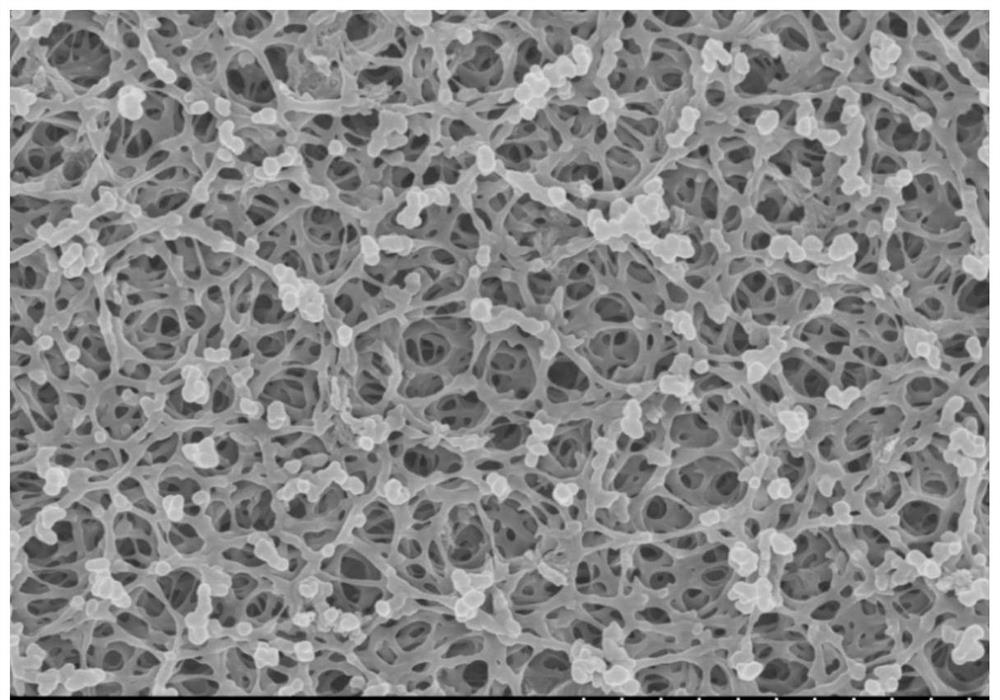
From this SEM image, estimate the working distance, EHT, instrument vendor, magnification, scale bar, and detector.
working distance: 8.8 mm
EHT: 5 kV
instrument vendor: Hitachi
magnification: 10 K X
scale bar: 5000 nm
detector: SE(M)